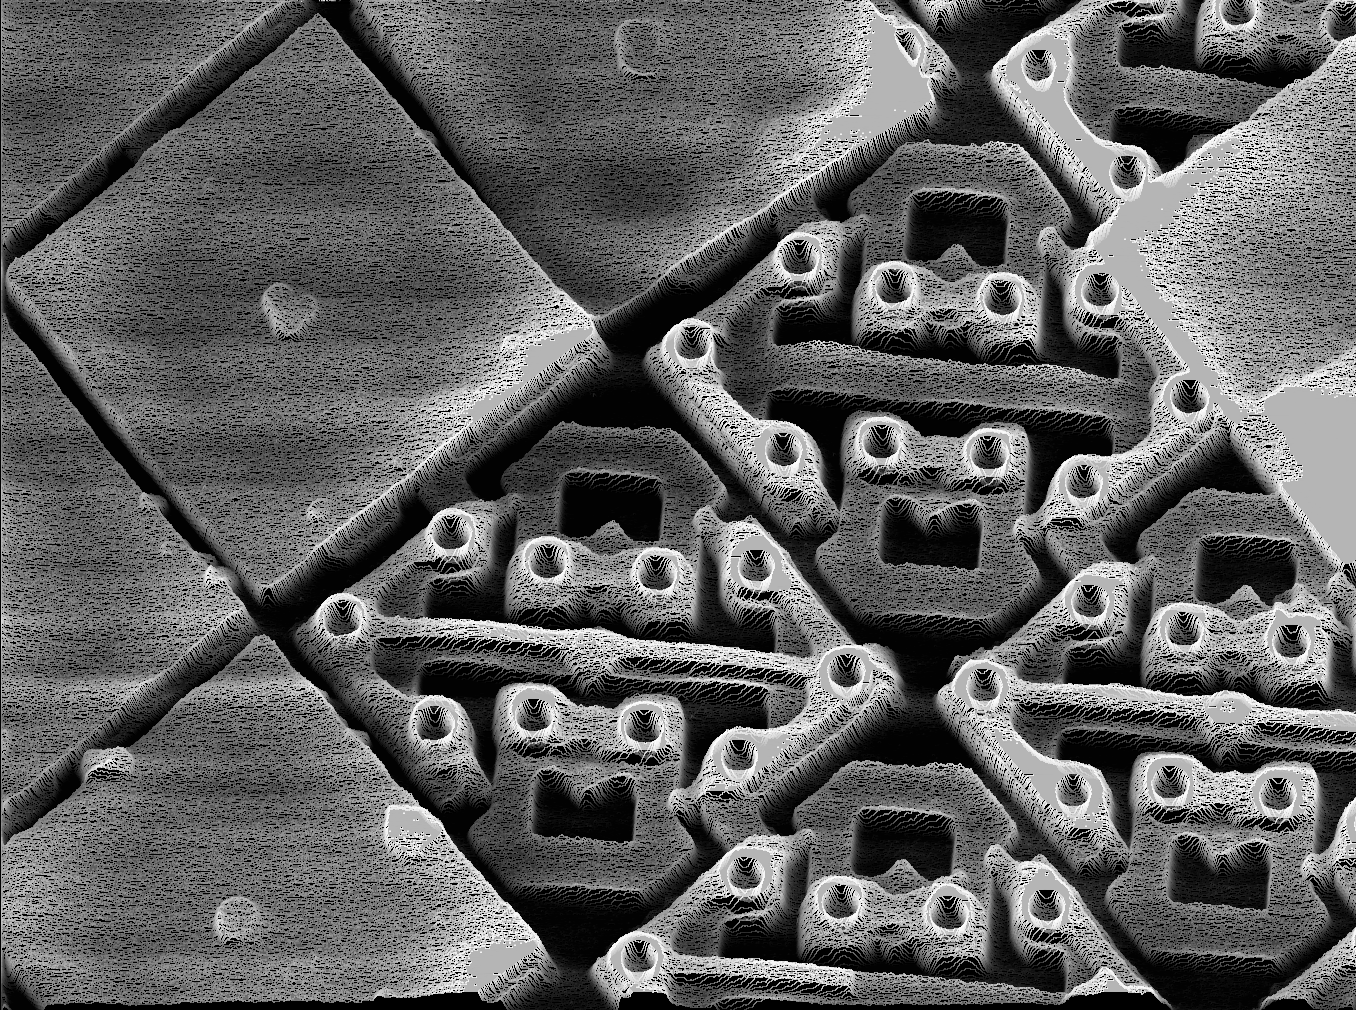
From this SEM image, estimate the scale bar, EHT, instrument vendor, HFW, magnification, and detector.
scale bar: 3330 nm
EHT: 15 kV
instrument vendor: other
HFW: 40 µm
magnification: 3 K X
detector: SE +Y-МОД